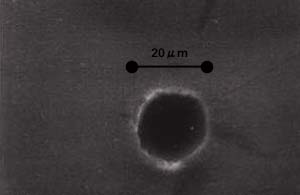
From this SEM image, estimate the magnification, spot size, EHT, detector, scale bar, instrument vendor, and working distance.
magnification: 1.002 K X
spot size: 3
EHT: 20 kV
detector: SE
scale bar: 20000 nm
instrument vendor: FEI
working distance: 9.6 mm